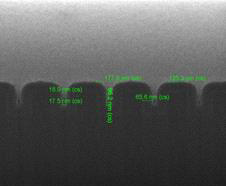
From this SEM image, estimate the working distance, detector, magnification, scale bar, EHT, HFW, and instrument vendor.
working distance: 10 mm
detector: ETD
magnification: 200 K X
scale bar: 500 nm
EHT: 5 kV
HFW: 1.49 µm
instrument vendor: FEI